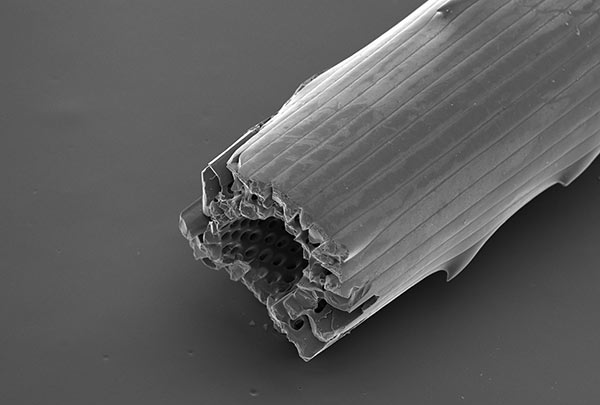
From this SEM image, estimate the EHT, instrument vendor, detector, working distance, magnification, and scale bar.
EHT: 15 kV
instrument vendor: Hitachi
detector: SE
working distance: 13.2 mm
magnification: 0.1 K X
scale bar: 500000 nm